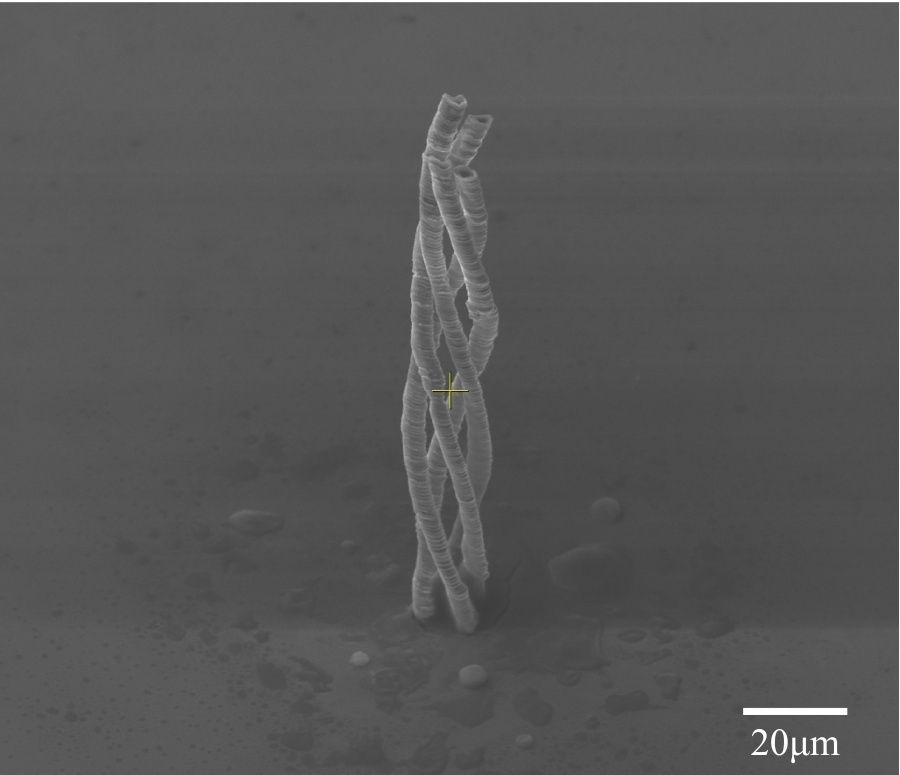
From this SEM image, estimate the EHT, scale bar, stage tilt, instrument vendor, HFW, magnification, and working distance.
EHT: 20 kV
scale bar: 50000 nm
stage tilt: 45°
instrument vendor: FEI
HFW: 173 µm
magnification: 1.565 K X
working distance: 15 mm